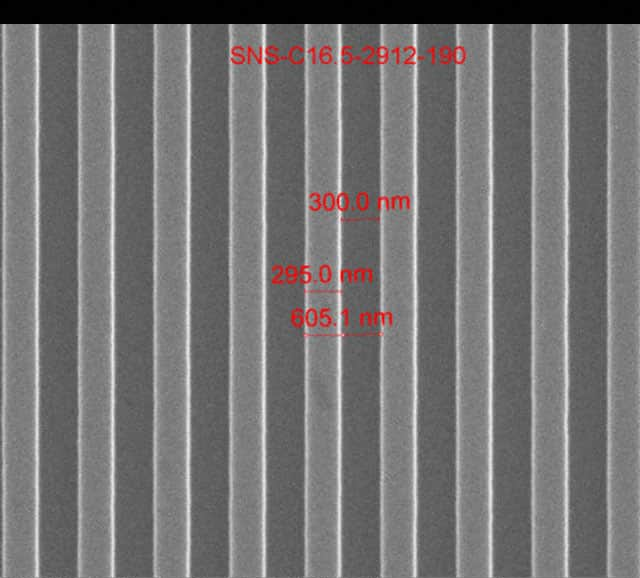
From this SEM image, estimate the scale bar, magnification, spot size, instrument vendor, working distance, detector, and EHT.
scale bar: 2000 nm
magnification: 50 K X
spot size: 1.5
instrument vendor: FEI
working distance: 10.7 mm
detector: ETD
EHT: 15 kV